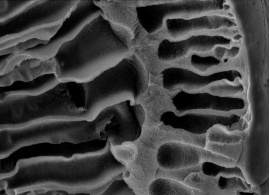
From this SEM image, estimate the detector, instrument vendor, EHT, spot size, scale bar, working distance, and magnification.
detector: SEI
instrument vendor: other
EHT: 20 kV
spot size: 1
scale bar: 10000 nm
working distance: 7.4 mm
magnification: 1 K X